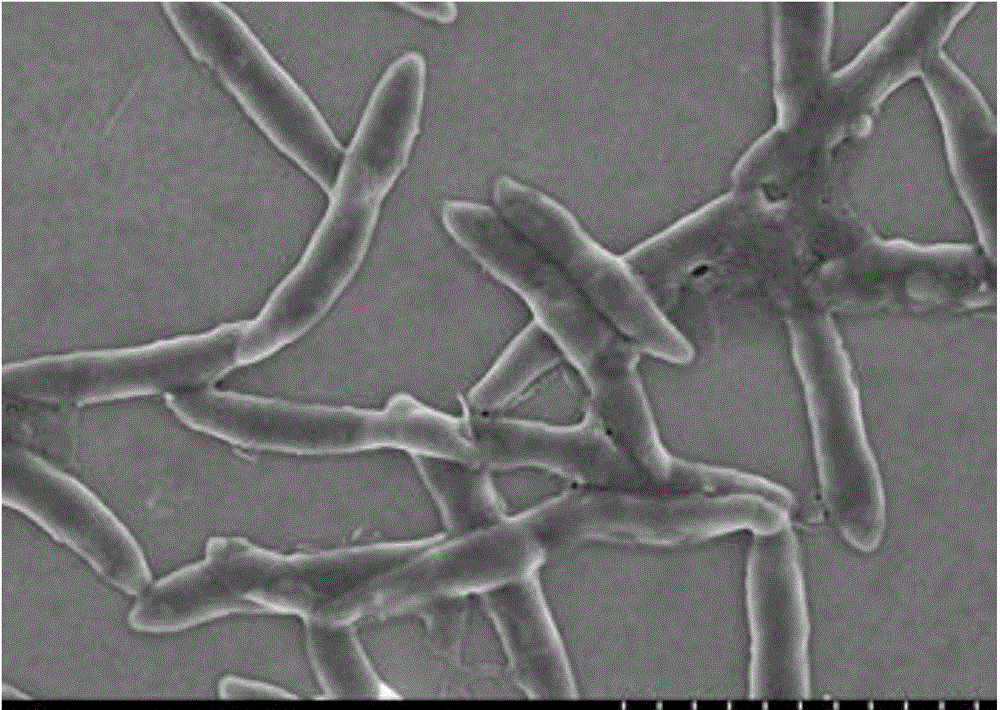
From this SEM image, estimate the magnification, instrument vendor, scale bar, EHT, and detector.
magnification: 15 K X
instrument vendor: Hitachi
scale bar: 3000 nm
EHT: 3 kV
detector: SE(M)